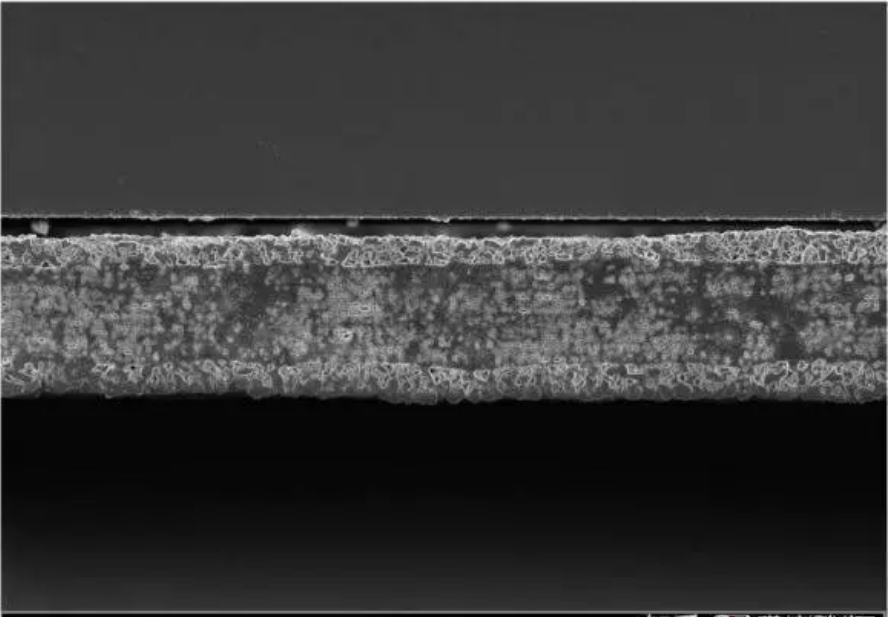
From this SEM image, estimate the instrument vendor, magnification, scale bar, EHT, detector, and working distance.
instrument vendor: Zeiss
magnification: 2 K X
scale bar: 30000 nm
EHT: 3 kV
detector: InLens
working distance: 2.3 mm